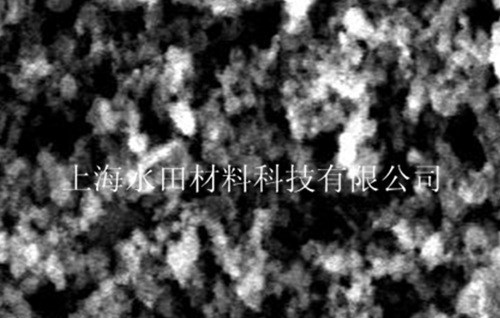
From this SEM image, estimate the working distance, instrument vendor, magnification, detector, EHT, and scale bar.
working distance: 9.7 mm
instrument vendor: FEI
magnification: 10 K X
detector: ETD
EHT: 20 kV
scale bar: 5000 nm